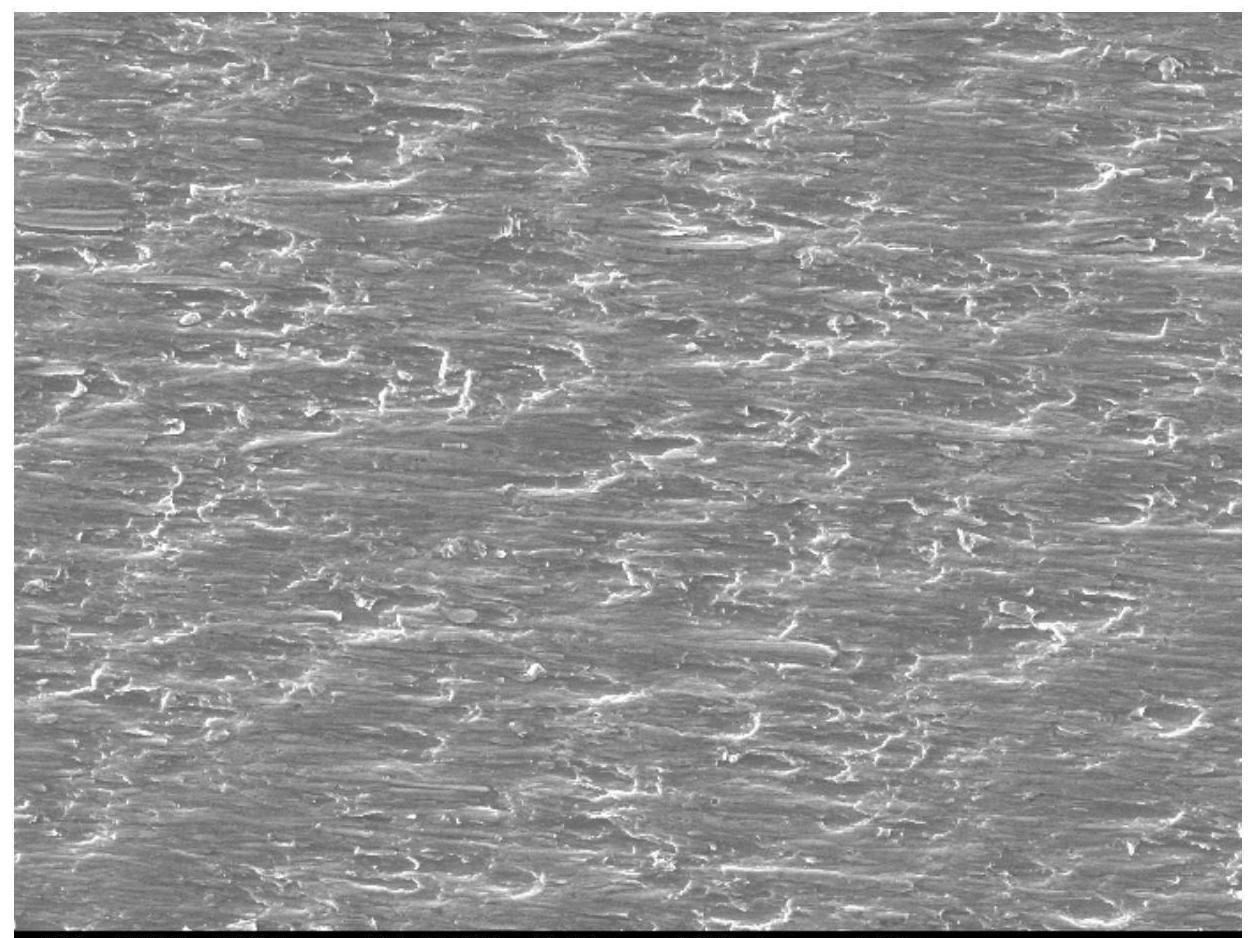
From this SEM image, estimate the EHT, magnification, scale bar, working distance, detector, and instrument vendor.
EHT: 15 kV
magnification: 1 K X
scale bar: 10000 nm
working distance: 9.4 mm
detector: SED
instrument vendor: JEOL